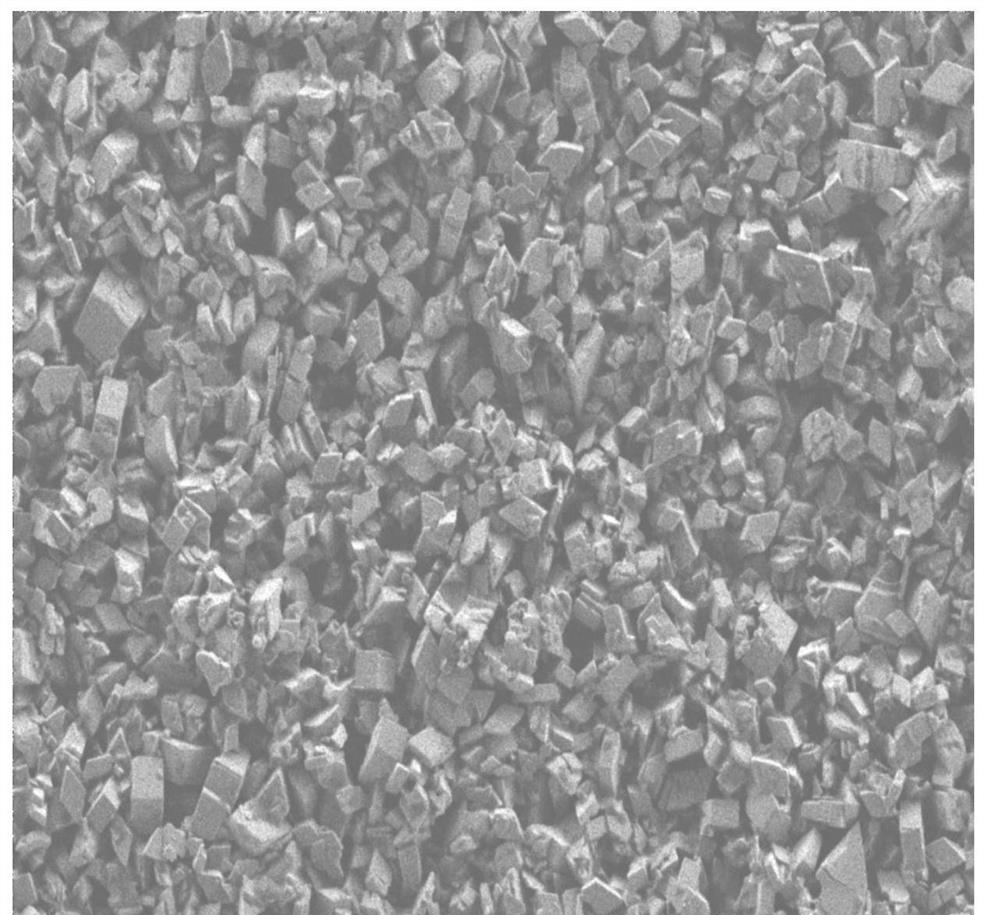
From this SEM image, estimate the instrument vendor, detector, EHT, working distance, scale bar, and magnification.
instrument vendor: Zeiss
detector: SE2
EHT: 1 kV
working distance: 3.5 mm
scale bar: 1000 nm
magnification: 10 K X